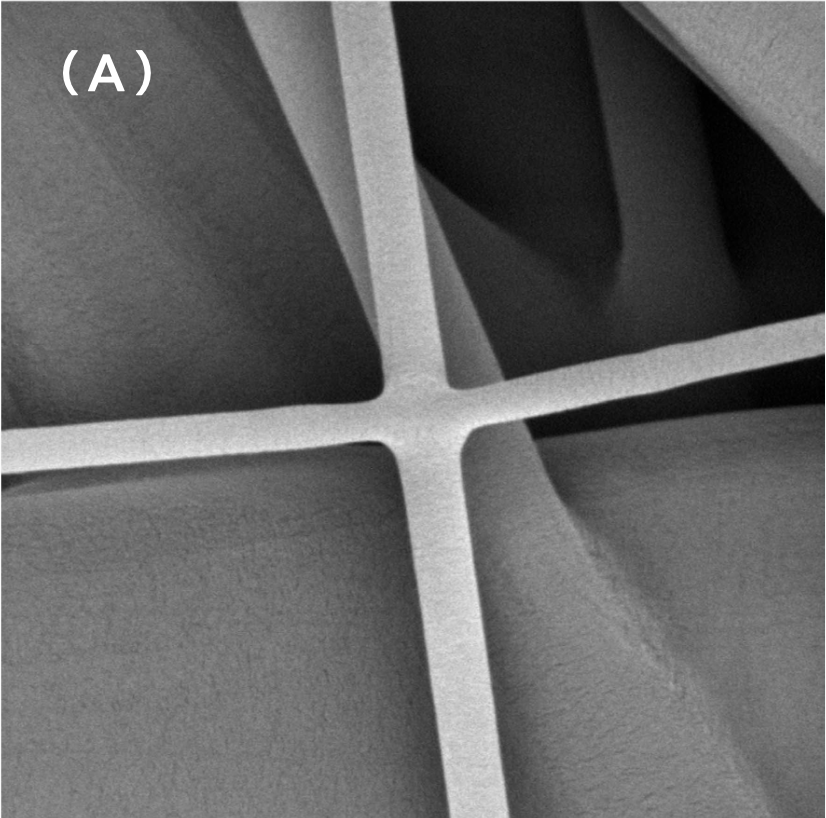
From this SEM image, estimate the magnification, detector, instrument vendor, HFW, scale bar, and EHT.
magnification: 20 K X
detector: BSD Full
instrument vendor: other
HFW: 13.4 µm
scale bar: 3000 nm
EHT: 10 kV